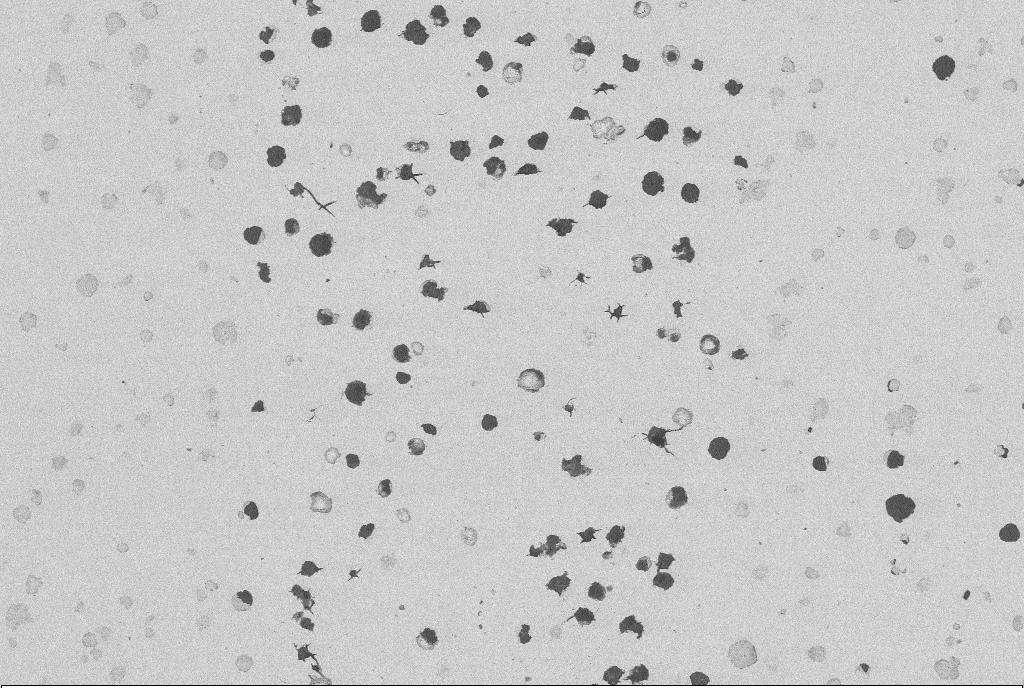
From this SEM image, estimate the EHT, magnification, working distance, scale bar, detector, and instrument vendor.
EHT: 20 kV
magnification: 0.2 K X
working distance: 13.5 mm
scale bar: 100000 nm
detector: NTS BSD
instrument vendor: Zeiss